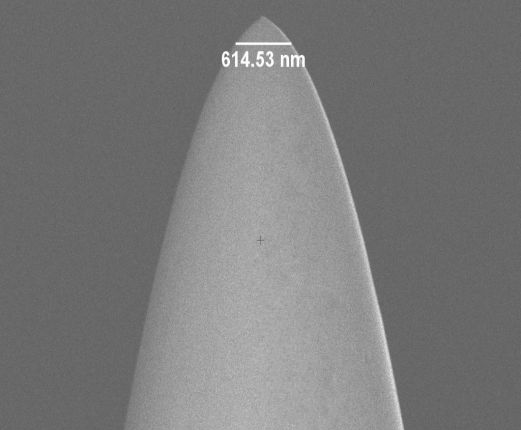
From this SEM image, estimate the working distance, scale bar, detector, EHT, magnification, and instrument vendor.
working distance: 4.6 mm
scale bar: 2000 nm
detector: InLens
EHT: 2 kV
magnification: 20 K X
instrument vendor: Zeiss